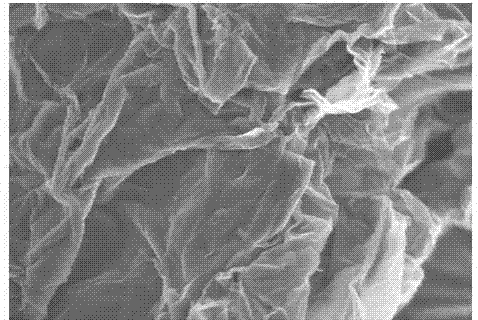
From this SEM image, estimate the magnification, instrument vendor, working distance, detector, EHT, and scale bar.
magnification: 9 K X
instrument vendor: Hitachi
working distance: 4 mm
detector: SE(U)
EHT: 5 kV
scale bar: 5000 nm